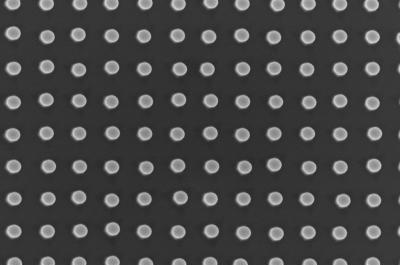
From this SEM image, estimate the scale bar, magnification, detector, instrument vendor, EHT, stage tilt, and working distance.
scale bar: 1000 nm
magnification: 50.1 K X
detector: InLens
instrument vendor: Zeiss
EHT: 10 kV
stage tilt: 2°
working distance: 6.8 mm